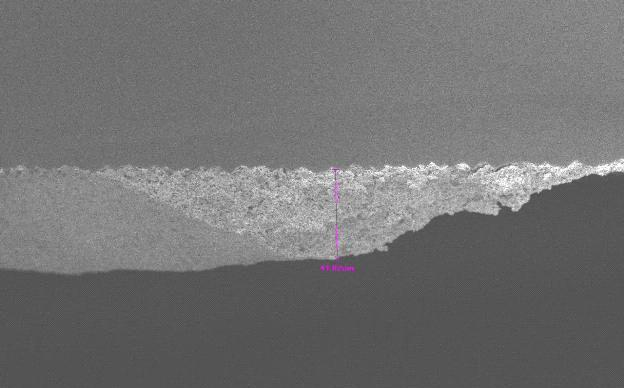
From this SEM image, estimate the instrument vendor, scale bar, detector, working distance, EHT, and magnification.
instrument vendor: JEOL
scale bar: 10000 nm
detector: SEI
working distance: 8.1 mm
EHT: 10 kV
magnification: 0.5 K X